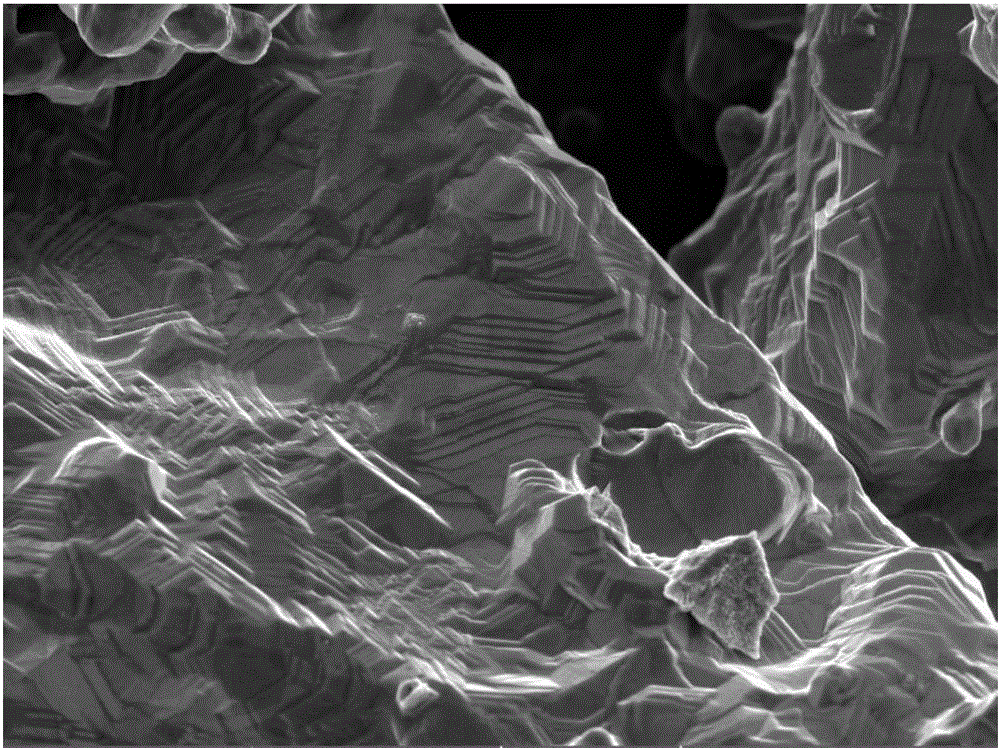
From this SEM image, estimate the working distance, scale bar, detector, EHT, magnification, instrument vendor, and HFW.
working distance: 4.98 mm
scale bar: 10000 nm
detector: InBeam SE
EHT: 10 kV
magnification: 5 K X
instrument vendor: Tescan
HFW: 55.4 µm